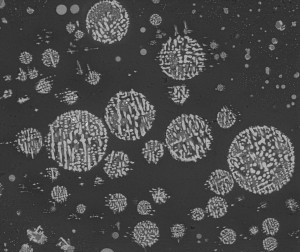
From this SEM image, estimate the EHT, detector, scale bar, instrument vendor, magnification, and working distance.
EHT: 20 kV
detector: BSED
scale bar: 100000 nm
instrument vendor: FEI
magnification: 1 K X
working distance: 9.8 mm